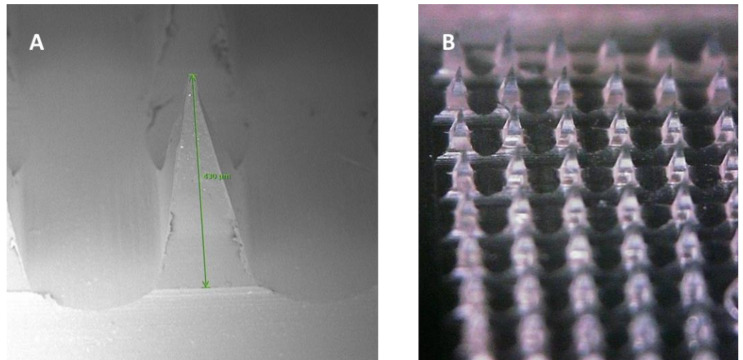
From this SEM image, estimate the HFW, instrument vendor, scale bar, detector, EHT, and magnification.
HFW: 753 µm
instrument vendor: other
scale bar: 200000 nm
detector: BSD Full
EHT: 5 kV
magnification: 0.32 K X